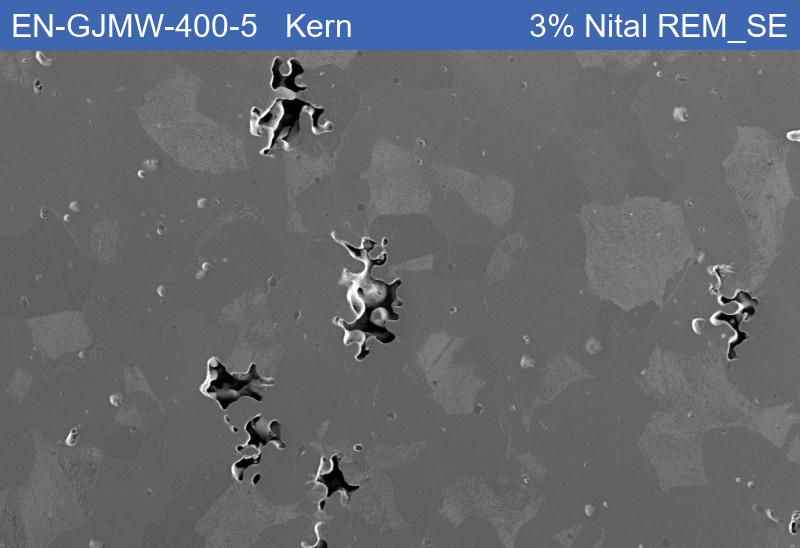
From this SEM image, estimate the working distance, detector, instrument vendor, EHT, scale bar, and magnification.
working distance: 8.52 mm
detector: SE1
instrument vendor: Zeiss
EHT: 15 kV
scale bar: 50000 nm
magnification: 0.2 K X